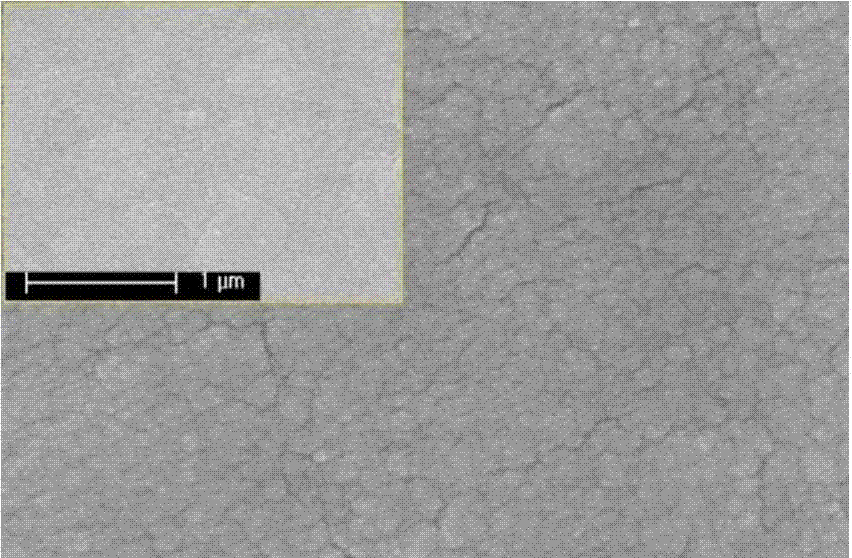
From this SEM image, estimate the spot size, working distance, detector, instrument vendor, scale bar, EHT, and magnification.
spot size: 3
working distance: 6.8 mm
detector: TLD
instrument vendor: FEI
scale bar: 200 nm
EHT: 20 kV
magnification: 200 K X